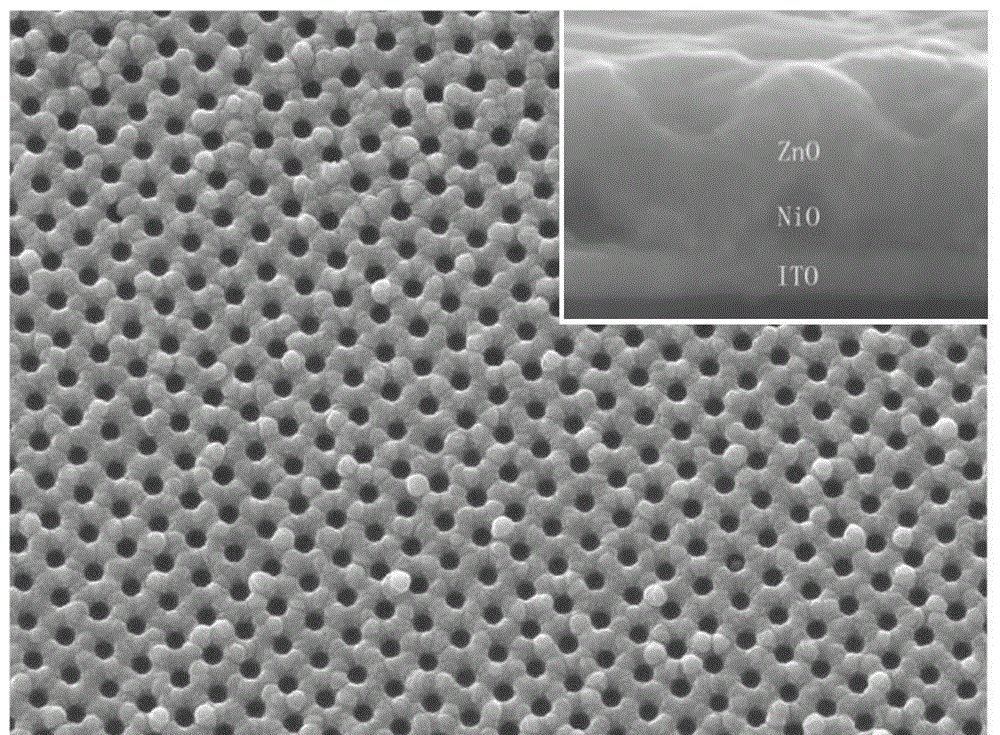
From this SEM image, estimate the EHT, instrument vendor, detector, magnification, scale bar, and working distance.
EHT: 15 kV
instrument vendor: JEOL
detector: LED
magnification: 10 K X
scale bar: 1000 nm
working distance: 6 mm